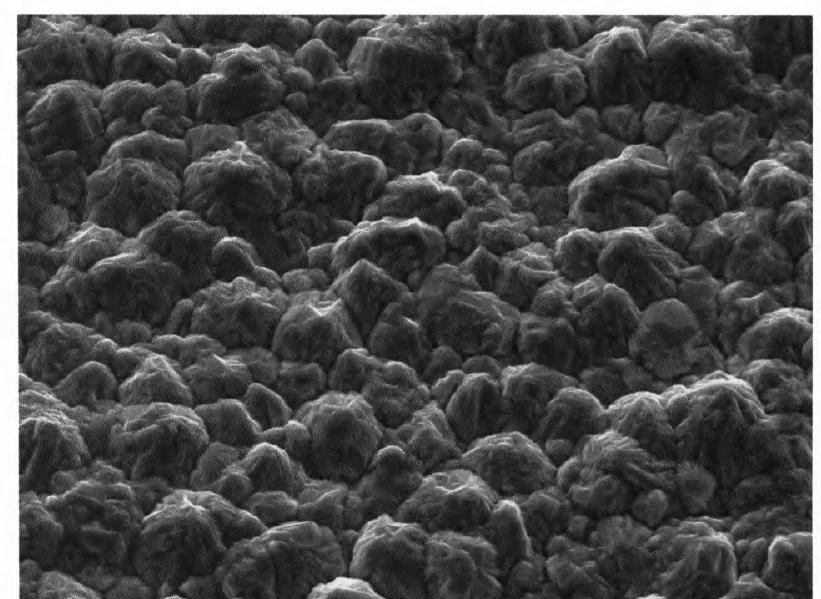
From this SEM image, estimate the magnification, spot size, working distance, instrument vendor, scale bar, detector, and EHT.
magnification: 2 K X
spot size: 0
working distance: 10.8 mm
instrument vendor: FEI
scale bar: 10000 nm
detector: SE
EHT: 20 kV